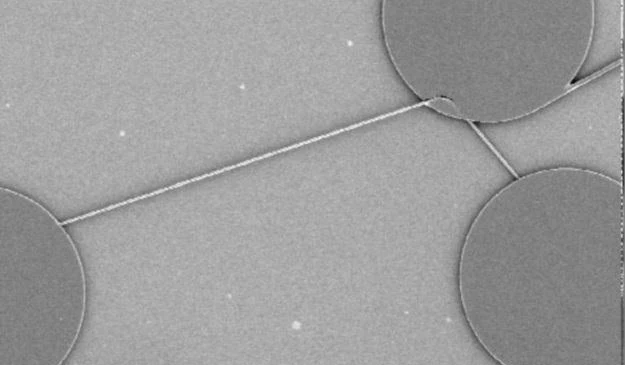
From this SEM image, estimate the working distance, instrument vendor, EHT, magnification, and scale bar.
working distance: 25 mm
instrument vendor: JEOL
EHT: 5 kV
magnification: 0.1 K X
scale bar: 100000 nm